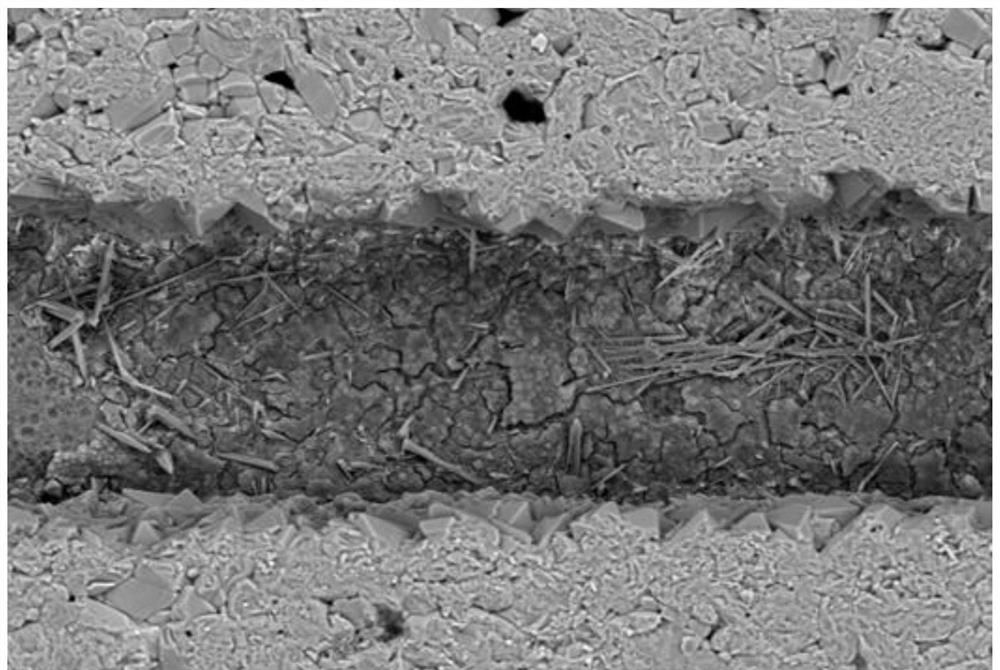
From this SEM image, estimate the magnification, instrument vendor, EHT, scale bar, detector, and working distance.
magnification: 0.3 K X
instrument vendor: Zeiss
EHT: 20 kV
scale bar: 40000 nm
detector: BSD1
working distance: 8.5 mm